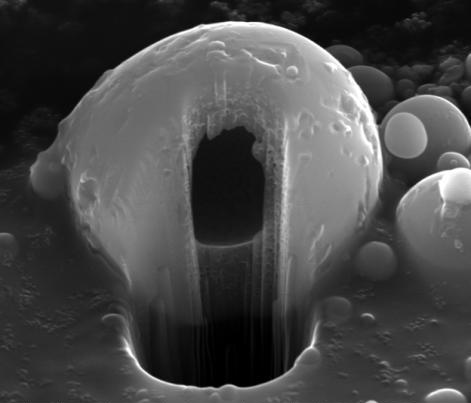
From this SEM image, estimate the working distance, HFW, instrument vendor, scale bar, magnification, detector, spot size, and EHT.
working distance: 10 mm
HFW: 12.4 µm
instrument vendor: FEI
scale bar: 3000 nm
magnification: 12 K X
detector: ETD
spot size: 6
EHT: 30 kV